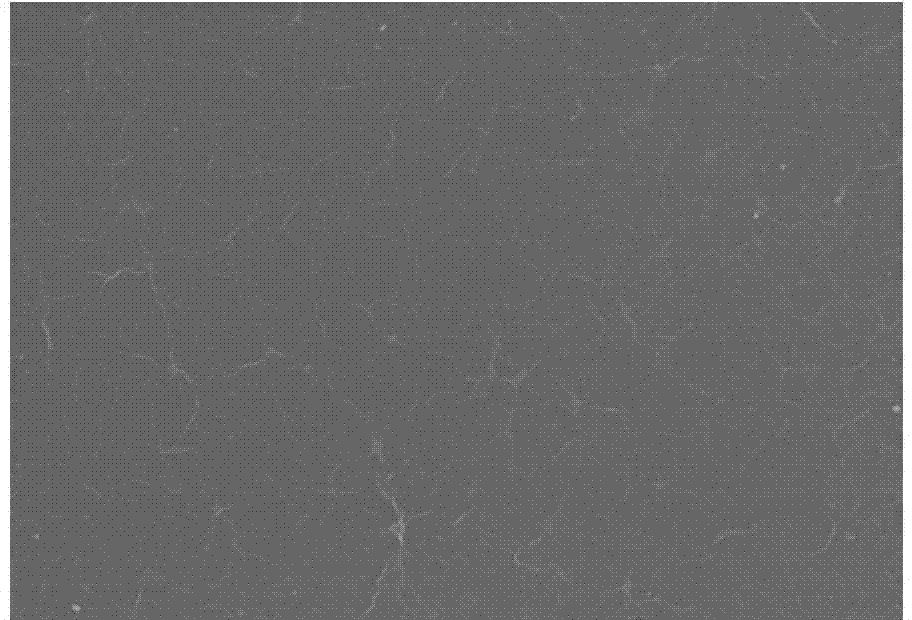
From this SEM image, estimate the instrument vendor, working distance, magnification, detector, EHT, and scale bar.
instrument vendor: Hitachi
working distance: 8.8 mm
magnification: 1 K X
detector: SE(M)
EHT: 5 kV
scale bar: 50000 nm